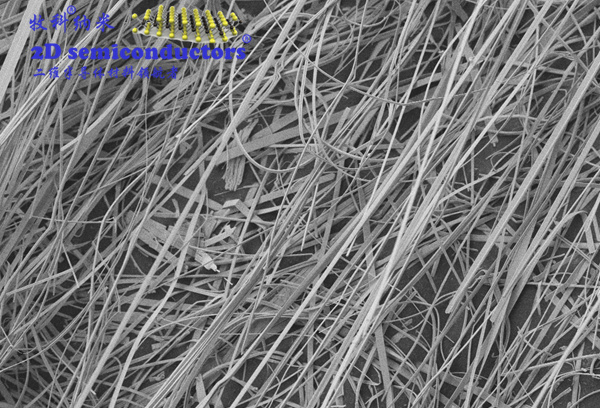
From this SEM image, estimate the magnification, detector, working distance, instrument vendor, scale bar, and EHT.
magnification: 1 K X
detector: SE2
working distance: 8.1 mm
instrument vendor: Zeiss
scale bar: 10000 nm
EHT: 5 kV